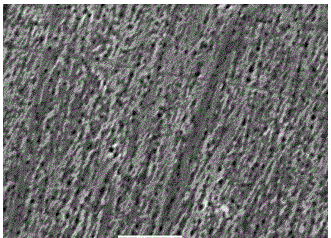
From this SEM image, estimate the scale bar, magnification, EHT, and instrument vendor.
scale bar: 5000 nm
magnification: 5 K X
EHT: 20 kV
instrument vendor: JEOL